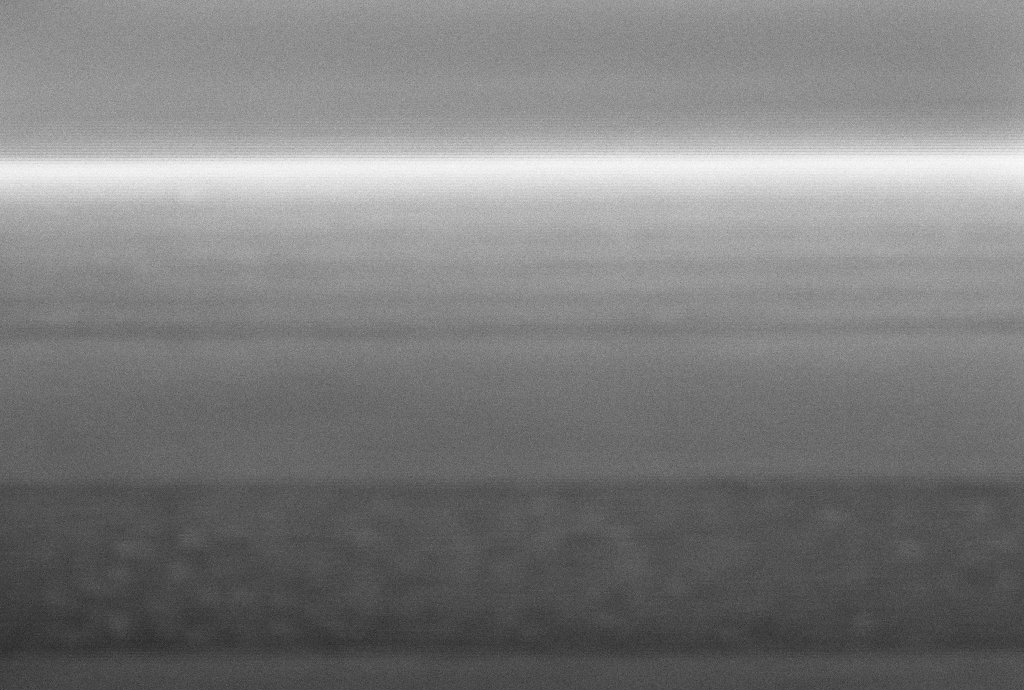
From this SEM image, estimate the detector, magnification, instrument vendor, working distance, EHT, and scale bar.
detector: InLens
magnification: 361.74 K X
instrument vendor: Zeiss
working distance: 5.4 mm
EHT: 5 kV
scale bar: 20 nm